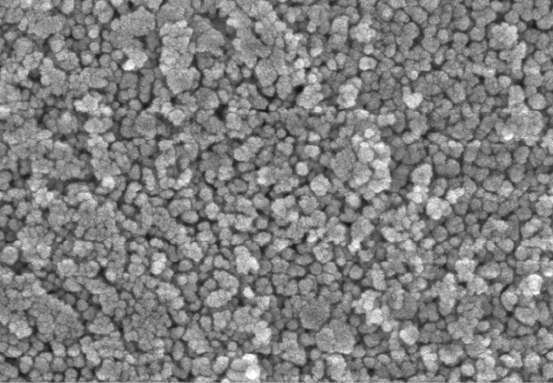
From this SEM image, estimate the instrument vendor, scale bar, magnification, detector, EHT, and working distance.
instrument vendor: Zeiss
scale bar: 100 nm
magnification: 100 K X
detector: InLens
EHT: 3 kV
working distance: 4 mm